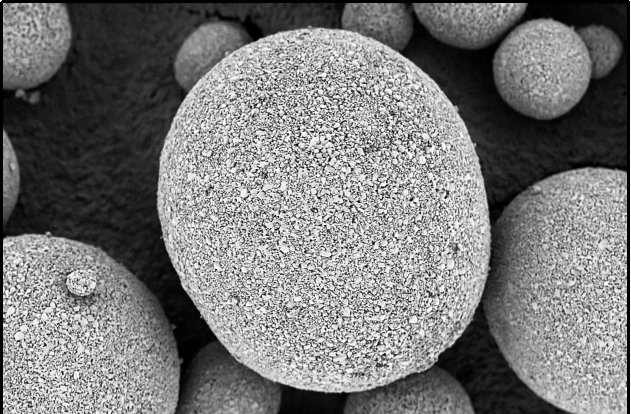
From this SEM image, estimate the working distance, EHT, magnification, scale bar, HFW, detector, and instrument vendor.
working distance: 3.3 mm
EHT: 1 kV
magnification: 1.5 K X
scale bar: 40000 nm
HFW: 84.7 µm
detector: T1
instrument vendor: Thermo